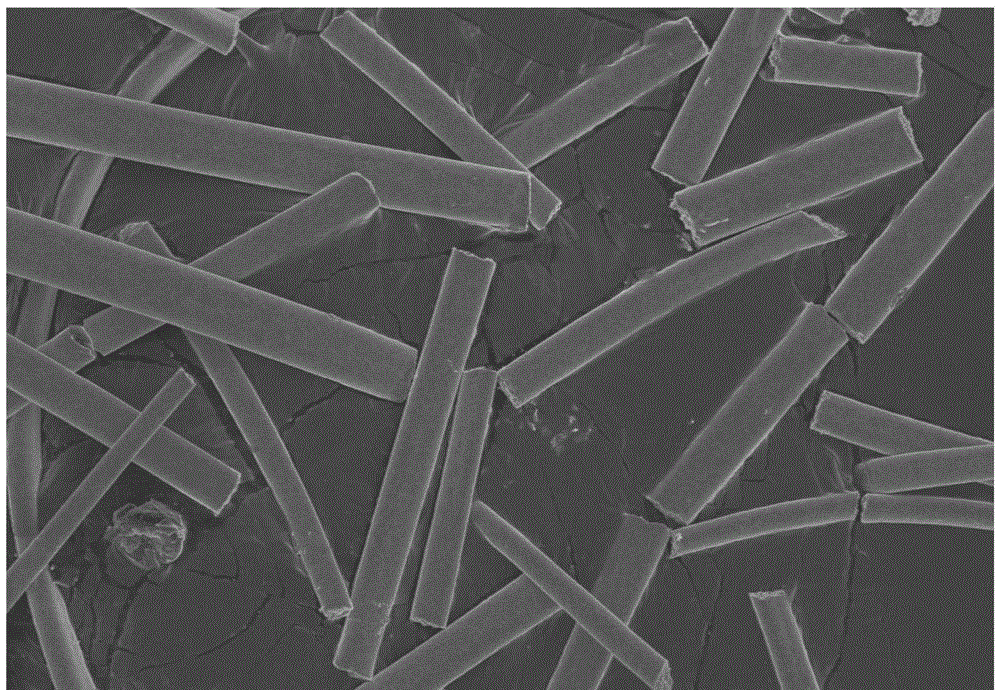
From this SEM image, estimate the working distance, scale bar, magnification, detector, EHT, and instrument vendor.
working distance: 9.9 mm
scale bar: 50000 nm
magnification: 1 K X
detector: SE(U)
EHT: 5 kV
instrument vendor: Hitachi